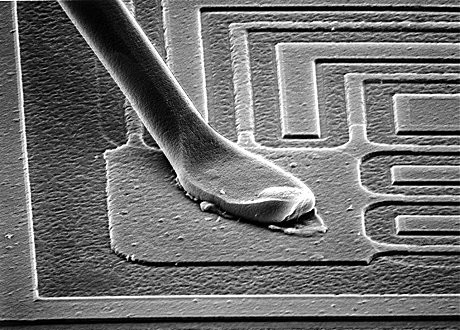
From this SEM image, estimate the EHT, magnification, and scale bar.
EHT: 30 kV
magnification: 0.5 K X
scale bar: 100000 nm